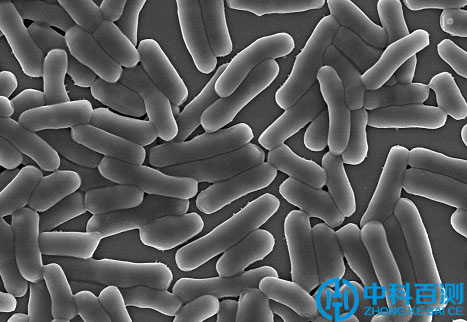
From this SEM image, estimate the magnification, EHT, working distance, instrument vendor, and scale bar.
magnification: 10 K X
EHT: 5 kV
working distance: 10.5 mm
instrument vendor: Hitachi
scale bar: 5000 nm